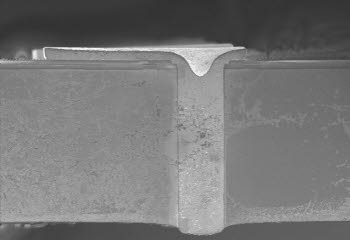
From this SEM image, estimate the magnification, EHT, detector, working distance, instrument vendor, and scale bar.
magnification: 0.5 K X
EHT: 1 kV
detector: SE(U)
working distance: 10.3 mm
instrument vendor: Hitachi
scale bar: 100000 nm